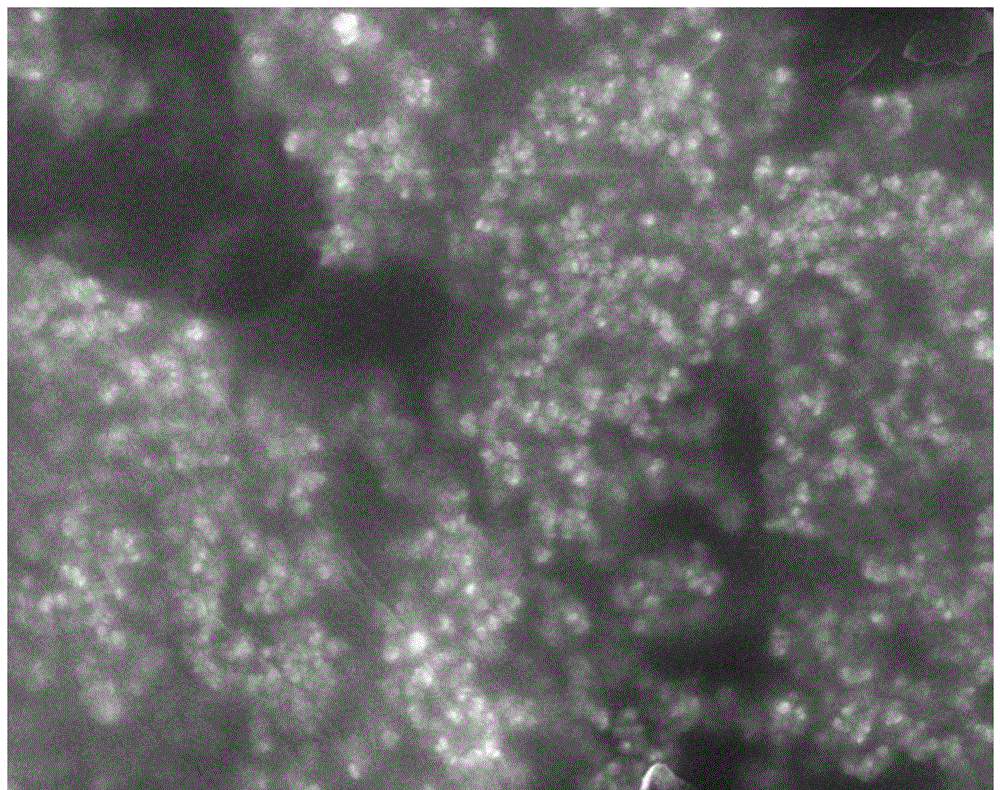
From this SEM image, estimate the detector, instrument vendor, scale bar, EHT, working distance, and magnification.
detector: SEI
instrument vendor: JEOL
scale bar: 1000 nm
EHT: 10 kV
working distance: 7.5 mm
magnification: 20 K X